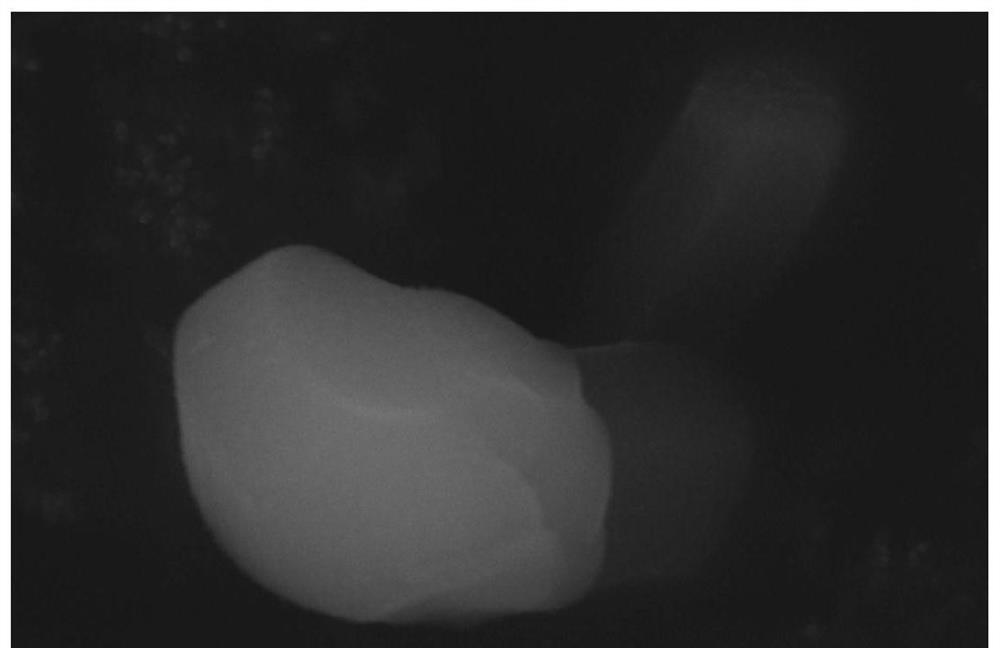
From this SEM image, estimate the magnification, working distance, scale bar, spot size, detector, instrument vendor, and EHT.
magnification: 20 K X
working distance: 7.5 mm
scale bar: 1000 nm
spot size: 3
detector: SE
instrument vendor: FEI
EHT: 15 kV